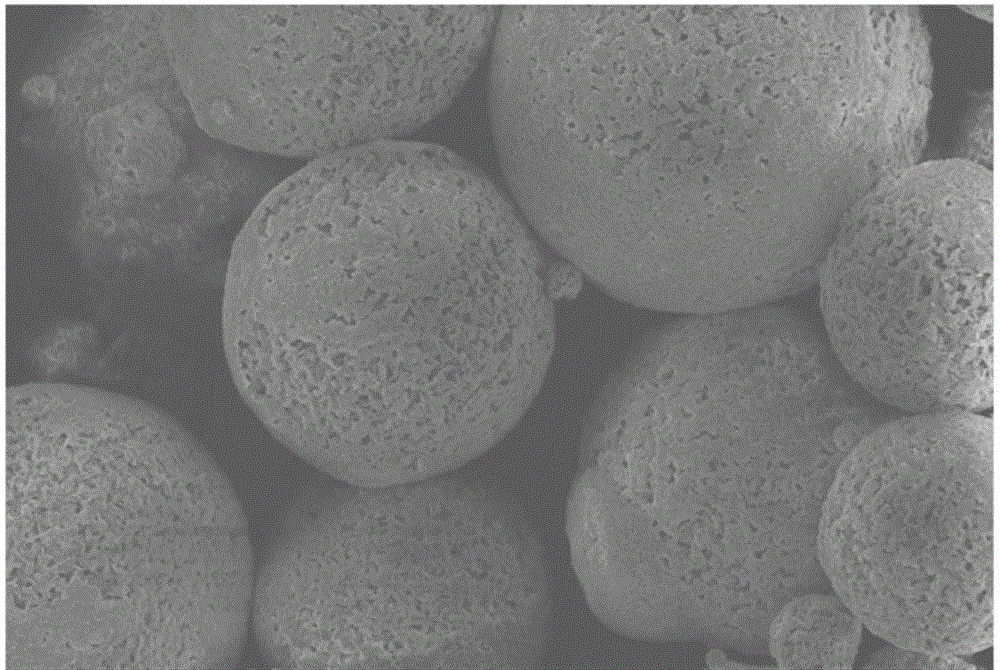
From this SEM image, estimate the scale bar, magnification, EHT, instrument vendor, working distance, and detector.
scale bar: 50000 nm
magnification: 0.7 K X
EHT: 5 kV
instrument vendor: Hitachi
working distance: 13.9 mm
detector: SE